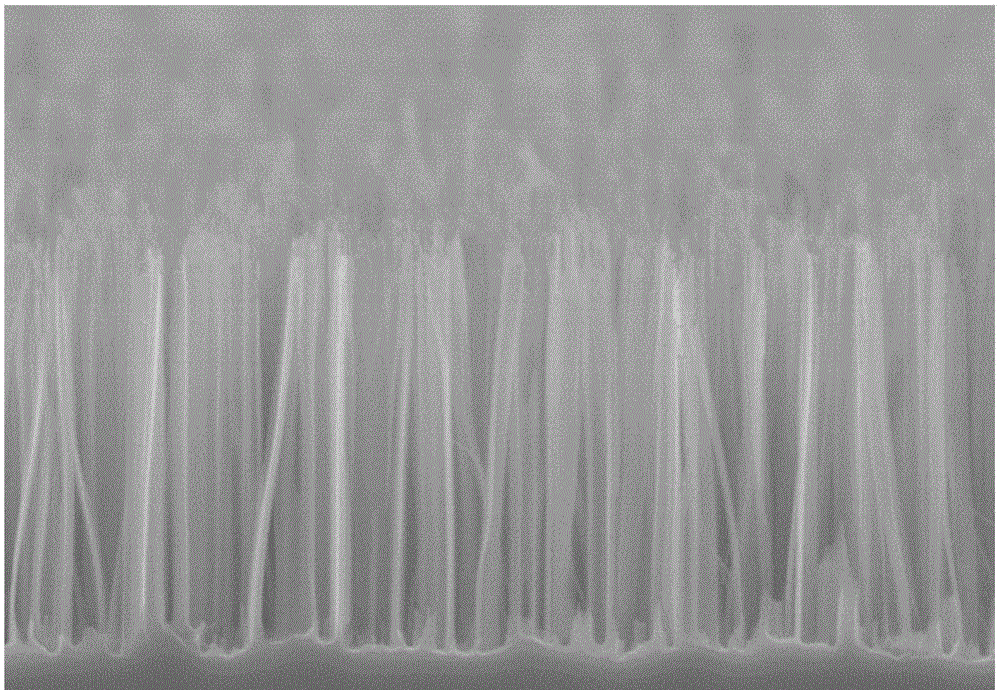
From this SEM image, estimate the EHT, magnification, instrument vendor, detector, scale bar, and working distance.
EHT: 5 kV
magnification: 22 K X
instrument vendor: Hitachi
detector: SE(M)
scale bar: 2000 nm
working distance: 8.3 mm